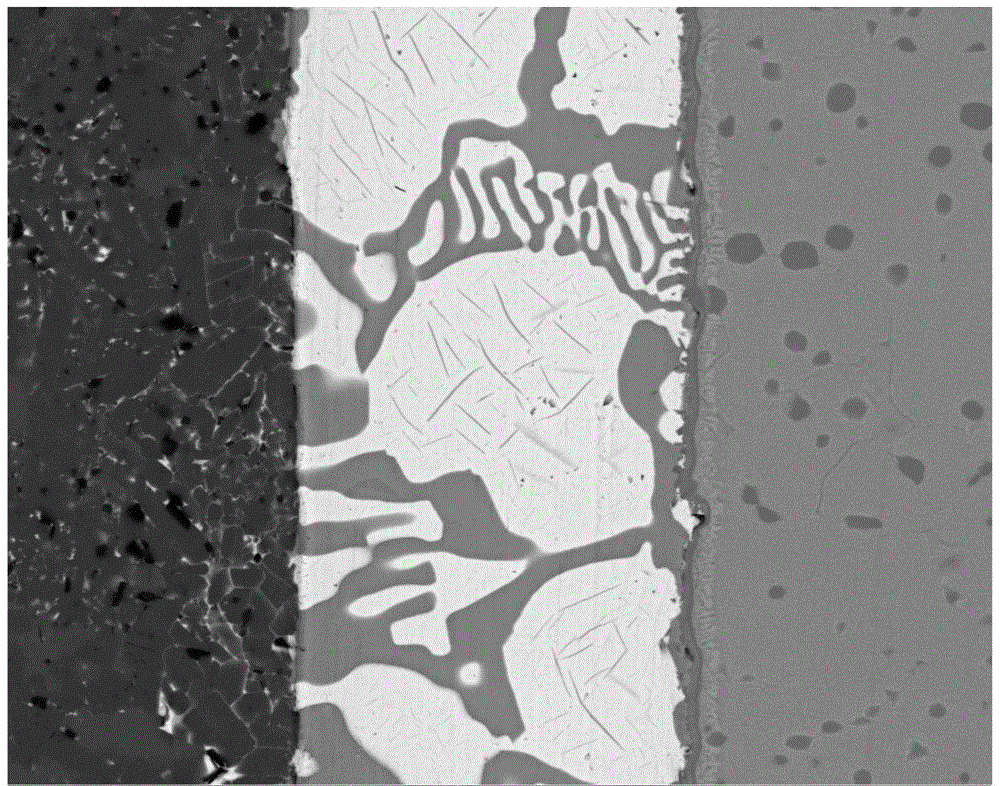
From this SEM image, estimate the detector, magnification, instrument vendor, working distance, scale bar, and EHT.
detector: Z Cont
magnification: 2.5 K X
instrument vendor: FEI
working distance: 13.2 mm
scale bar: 50000 nm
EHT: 30 kV